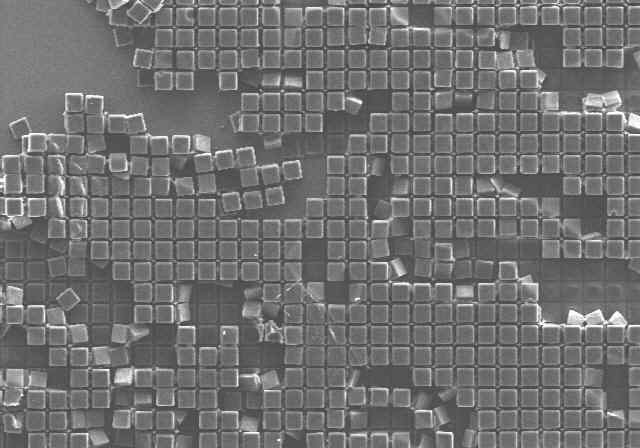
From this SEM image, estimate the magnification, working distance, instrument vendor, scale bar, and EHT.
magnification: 0.07 K X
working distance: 10.5 mm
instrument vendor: Hitachi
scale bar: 500000 nm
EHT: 25 kV